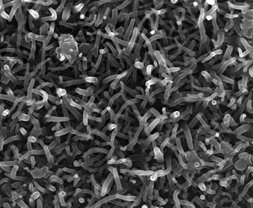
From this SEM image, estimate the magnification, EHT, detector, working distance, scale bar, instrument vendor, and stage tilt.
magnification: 25.026 K X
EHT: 10 kV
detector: TLD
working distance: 3.6 mm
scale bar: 2000 nm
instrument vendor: FEI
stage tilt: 0°